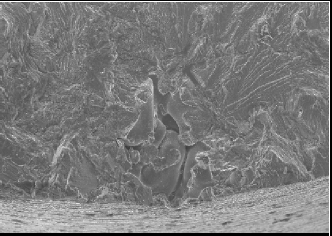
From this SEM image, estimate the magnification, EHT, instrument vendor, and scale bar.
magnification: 0.1 K X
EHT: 20 kV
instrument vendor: JEOL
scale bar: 500000 nm